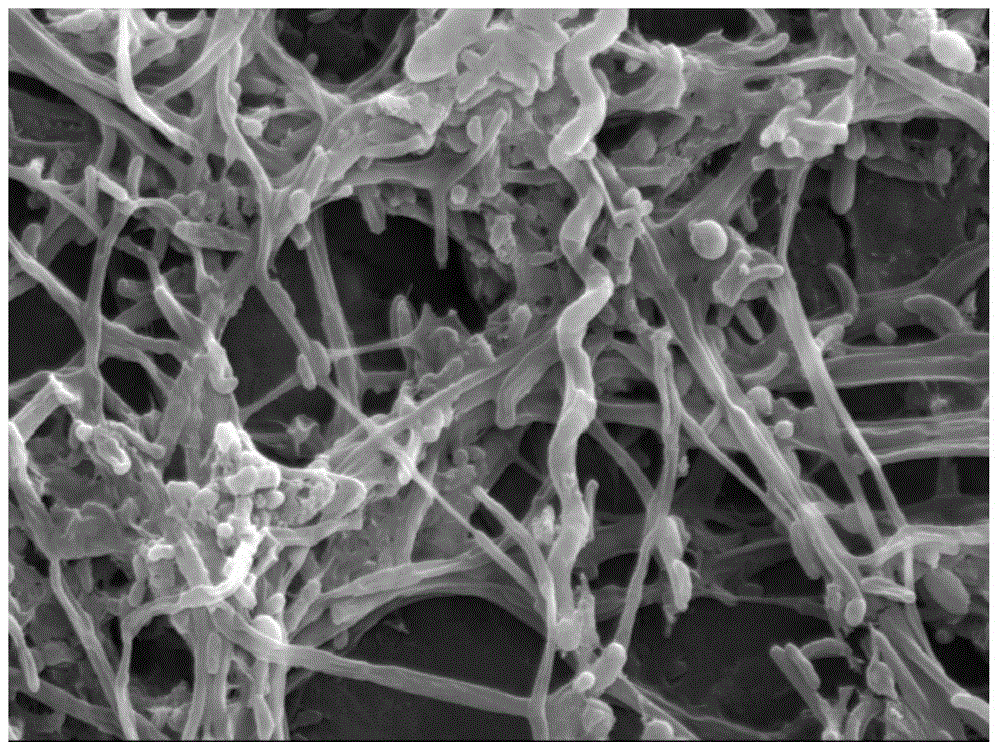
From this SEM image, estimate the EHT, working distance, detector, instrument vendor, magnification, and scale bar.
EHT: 15 kV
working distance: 18.6 mm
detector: SE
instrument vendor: Hitachi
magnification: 6 K X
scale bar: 5000 nm